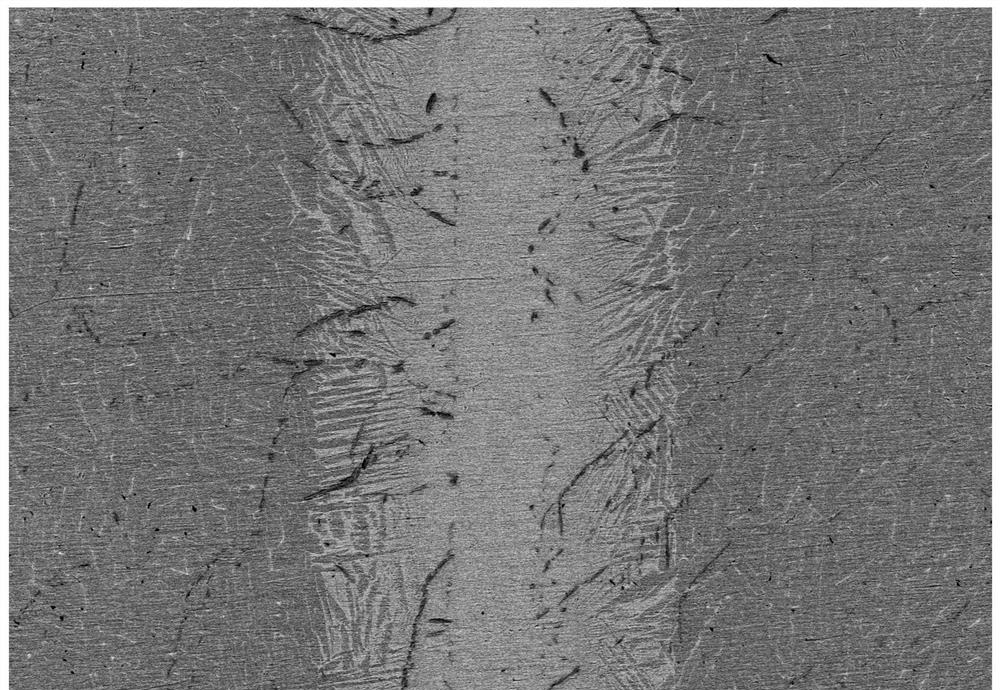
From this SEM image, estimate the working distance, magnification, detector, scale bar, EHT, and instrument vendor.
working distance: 8 mm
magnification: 0.2 K X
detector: PDBSE(CP)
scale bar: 200000 nm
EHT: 15 kV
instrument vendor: Hitachi